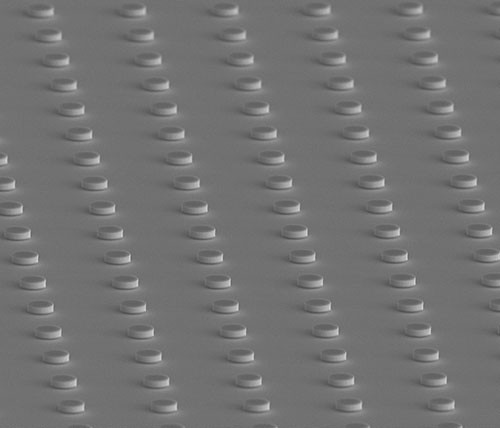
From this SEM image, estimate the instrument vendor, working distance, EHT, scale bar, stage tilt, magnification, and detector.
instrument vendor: FEI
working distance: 9.9 mm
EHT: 10 kV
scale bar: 30000 nm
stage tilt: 30°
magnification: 5 K X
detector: TLD